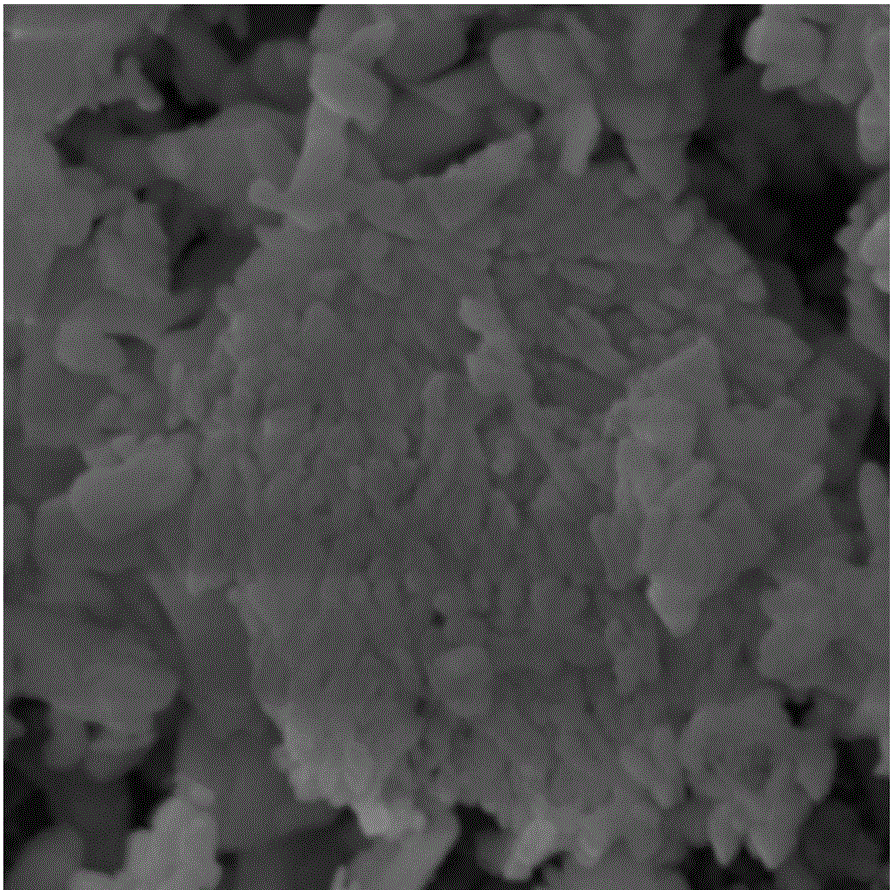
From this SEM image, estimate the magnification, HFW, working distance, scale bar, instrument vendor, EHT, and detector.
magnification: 20 K X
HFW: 7.52 µm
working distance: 18 mm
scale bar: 2000 nm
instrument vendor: Tescan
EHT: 20 kV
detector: SE